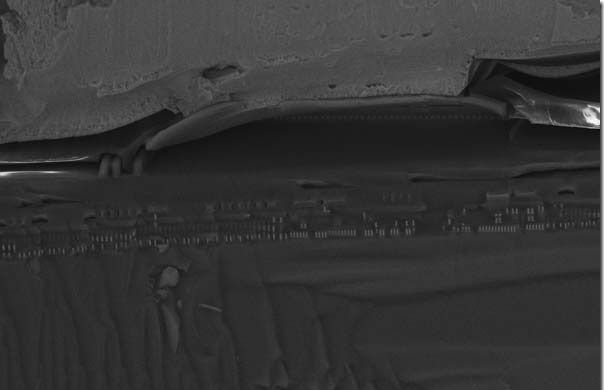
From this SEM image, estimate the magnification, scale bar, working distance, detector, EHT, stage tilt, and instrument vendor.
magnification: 3.16 K X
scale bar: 10000 nm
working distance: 4 mm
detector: InLens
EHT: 15 kV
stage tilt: -15°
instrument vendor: Zeiss